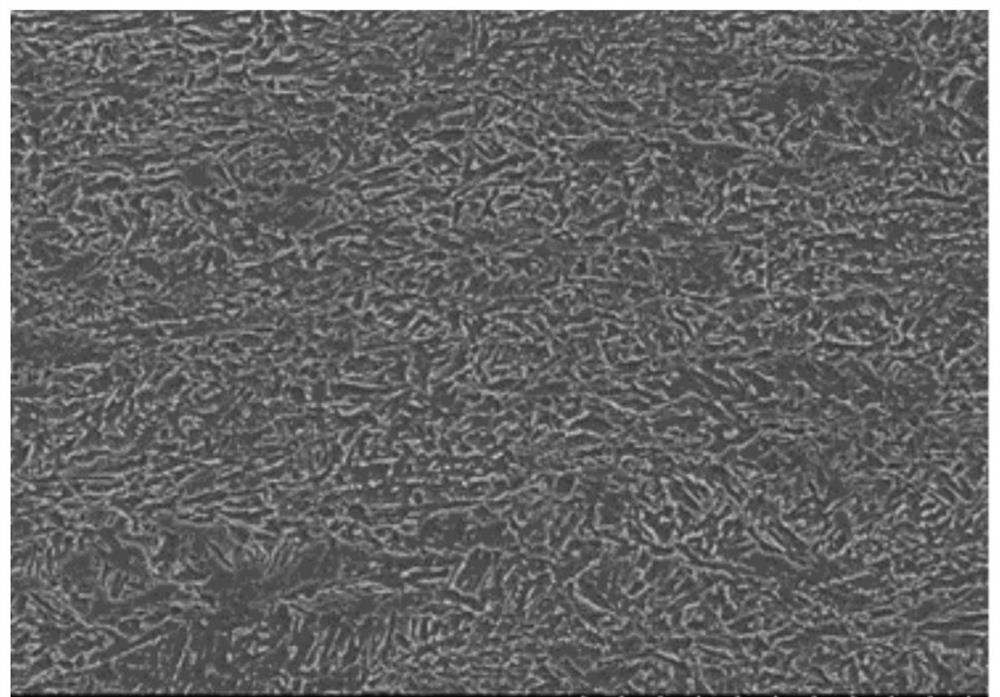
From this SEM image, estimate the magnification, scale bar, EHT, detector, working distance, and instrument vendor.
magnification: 1 K X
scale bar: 50000 nm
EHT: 15 kV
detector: SE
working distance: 6.9 mm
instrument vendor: Hitachi